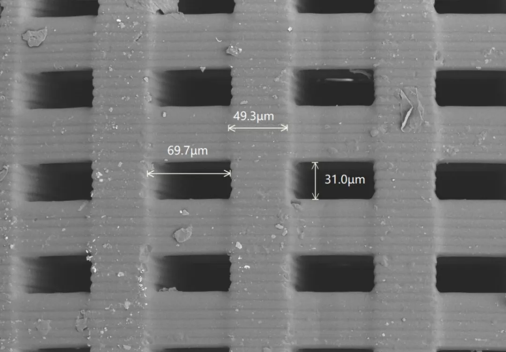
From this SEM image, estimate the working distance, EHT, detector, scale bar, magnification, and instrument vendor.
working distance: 10.9 mm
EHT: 10 kV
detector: BSE-ALL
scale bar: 100000 nm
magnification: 0.3 K X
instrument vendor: Hitachi